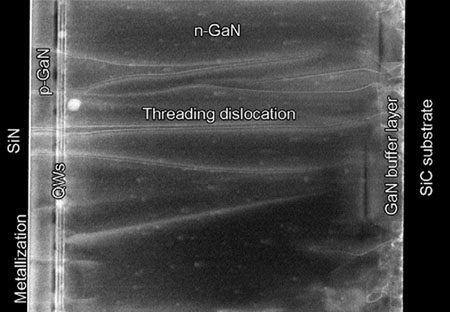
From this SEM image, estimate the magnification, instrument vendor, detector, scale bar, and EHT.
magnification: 45 K X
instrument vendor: Hitachi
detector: ZC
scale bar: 600 nm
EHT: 200 kV